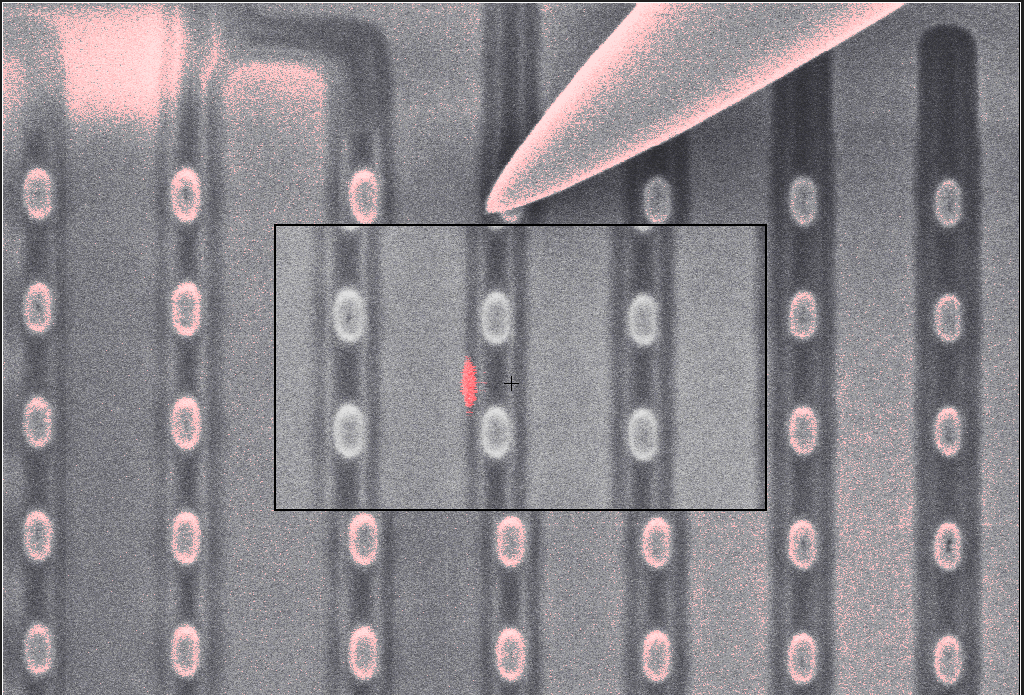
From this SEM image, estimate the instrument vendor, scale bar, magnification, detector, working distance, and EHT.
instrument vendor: Zeiss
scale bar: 1000 nm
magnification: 43.62 K X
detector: InLens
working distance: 4.6 mm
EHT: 3 kV